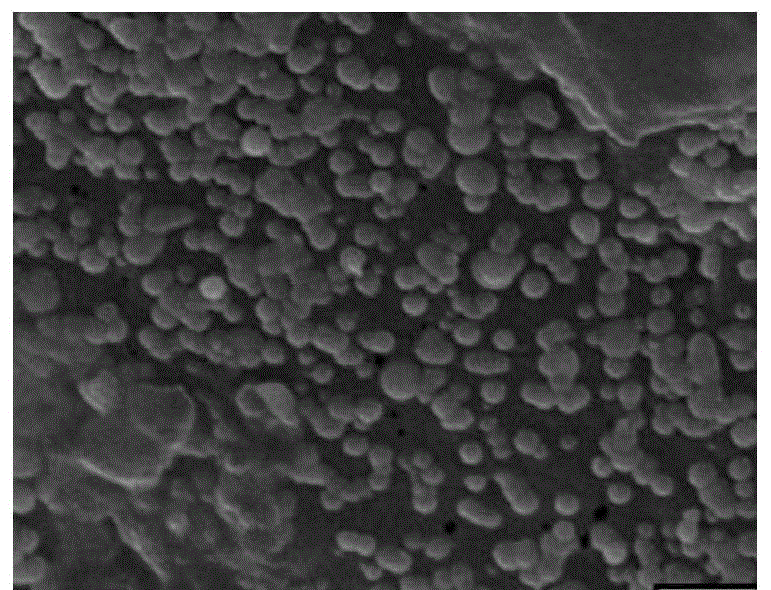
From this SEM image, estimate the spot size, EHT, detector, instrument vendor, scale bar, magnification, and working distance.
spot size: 33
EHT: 20 kV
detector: SEI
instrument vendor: JEOL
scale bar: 2000 nm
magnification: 9.5 K X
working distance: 12 mm